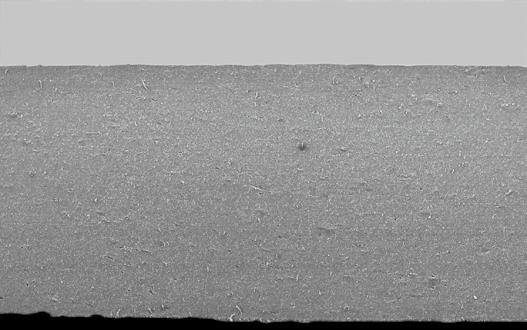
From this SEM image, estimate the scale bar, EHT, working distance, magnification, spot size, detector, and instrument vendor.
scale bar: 1000000 nm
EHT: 16 kV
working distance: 27.3 mm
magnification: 0.03 K X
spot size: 5.5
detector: SE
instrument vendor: FEI